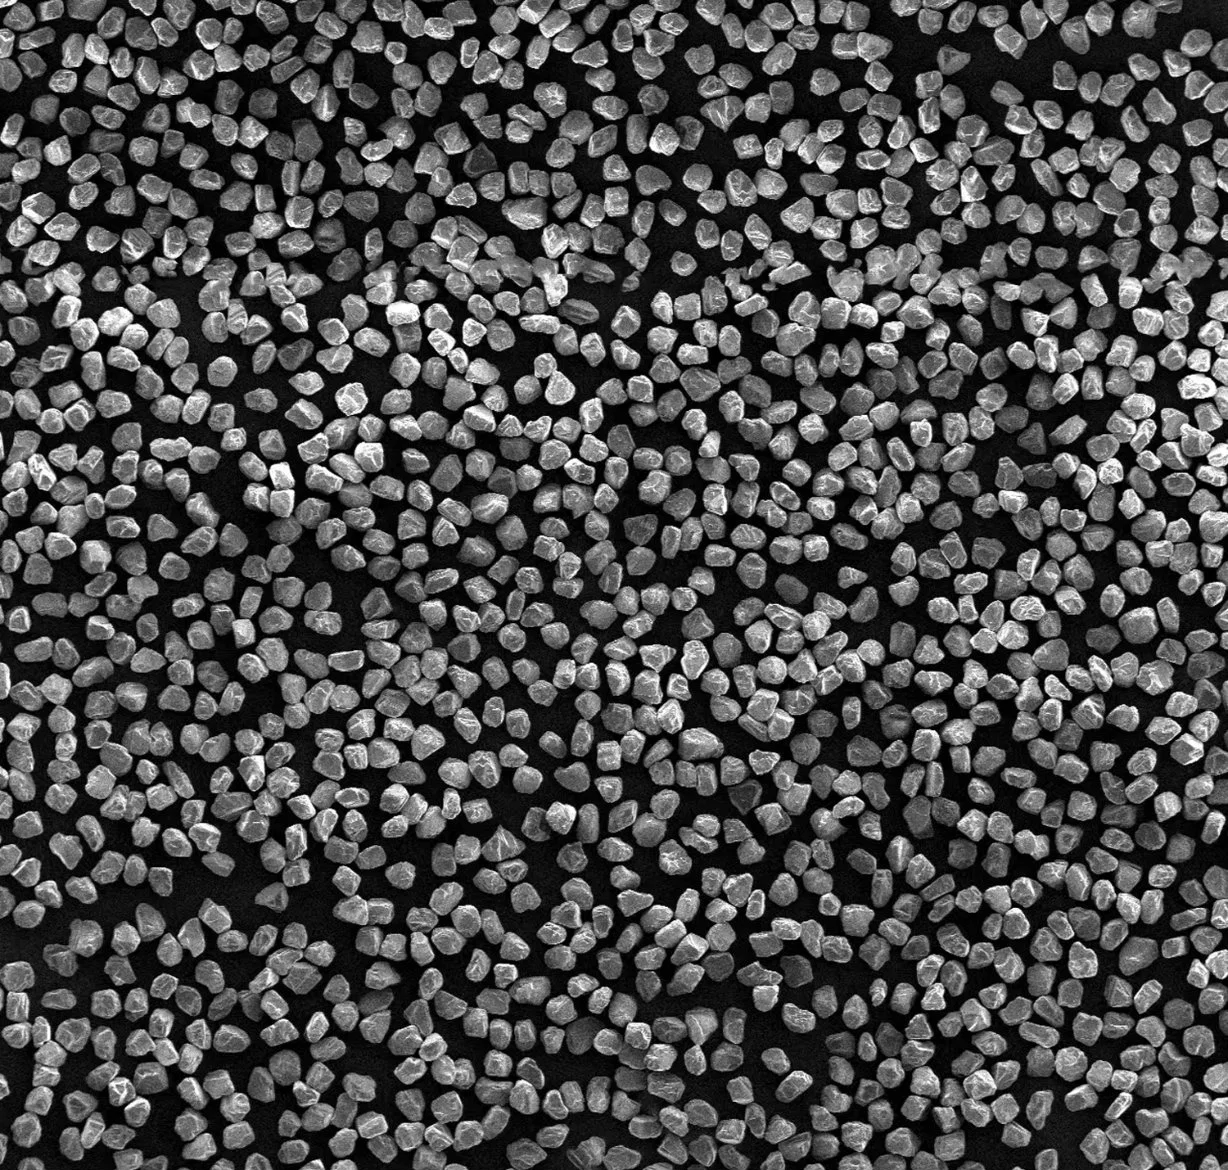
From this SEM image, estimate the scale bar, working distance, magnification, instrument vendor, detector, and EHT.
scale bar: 100000 nm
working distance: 10 mm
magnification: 0.1 K X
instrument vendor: JEOL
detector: SEI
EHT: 5 kV